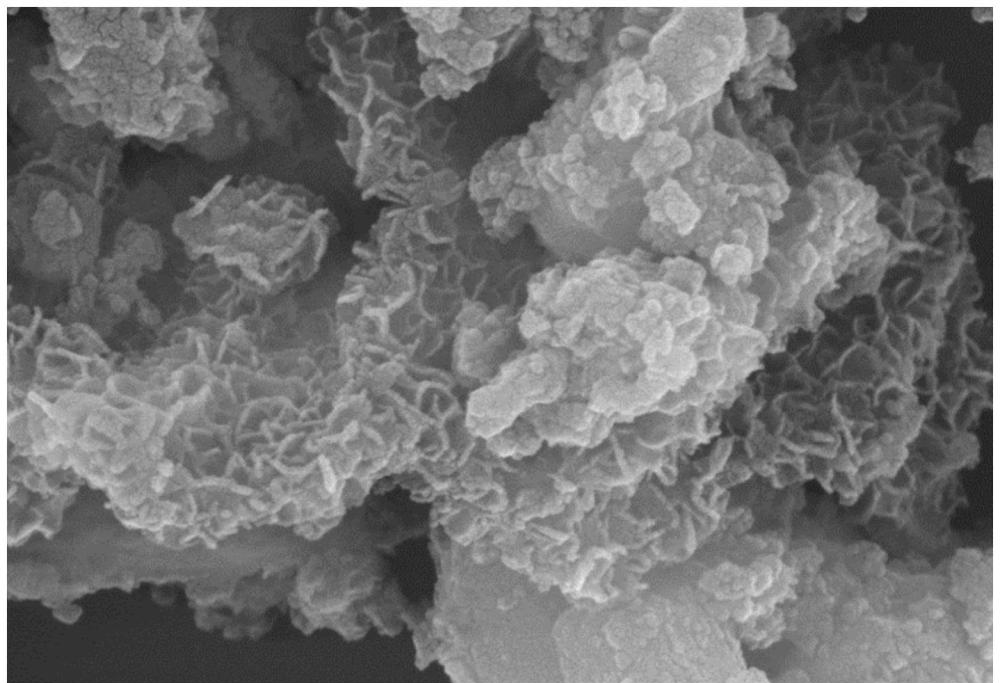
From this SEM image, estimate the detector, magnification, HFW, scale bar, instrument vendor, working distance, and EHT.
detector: In-Beam SE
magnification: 110 K X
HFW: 2.54 µm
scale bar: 200 nm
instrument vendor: Tescan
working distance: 6 mm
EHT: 15 kV